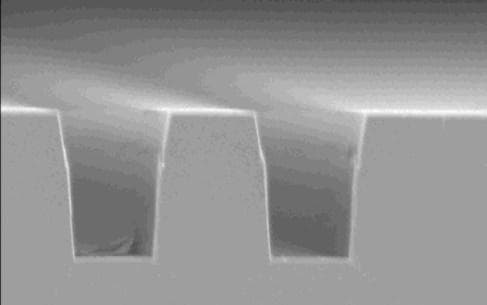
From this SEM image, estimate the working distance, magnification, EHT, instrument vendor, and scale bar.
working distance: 39 mm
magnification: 5 K X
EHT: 5 kV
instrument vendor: JEOL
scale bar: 1000 nm